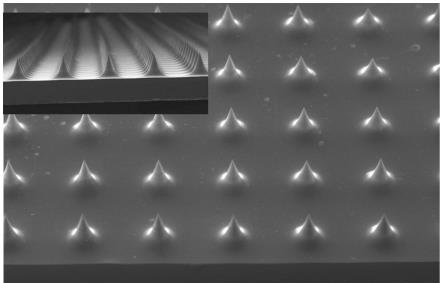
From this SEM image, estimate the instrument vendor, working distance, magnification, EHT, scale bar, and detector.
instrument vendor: Zeiss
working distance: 17 mm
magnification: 0.024 K X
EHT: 10 kV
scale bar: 200000 nm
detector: SE1